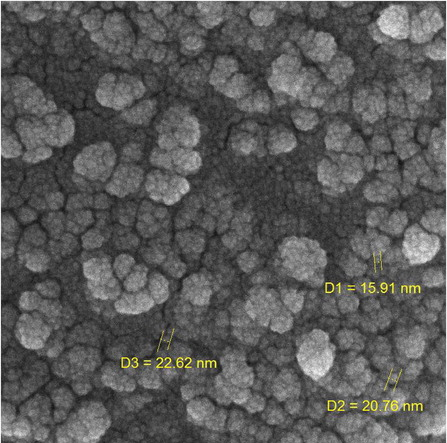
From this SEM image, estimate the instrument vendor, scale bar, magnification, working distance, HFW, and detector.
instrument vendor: Tescan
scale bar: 200 nm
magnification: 200 K X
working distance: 4.96 mm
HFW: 1.04 µm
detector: InBeam